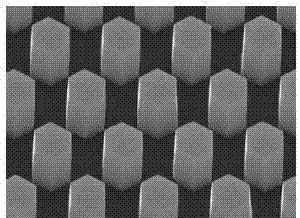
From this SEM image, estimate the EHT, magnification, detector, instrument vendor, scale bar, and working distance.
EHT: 15 kV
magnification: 20 K X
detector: SEI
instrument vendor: JEOL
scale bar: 1000 nm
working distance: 10 mm